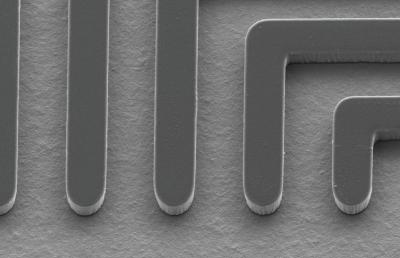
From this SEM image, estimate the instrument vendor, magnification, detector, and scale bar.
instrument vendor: Hitachi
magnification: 0.2 K X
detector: LM(L)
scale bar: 20000 nm